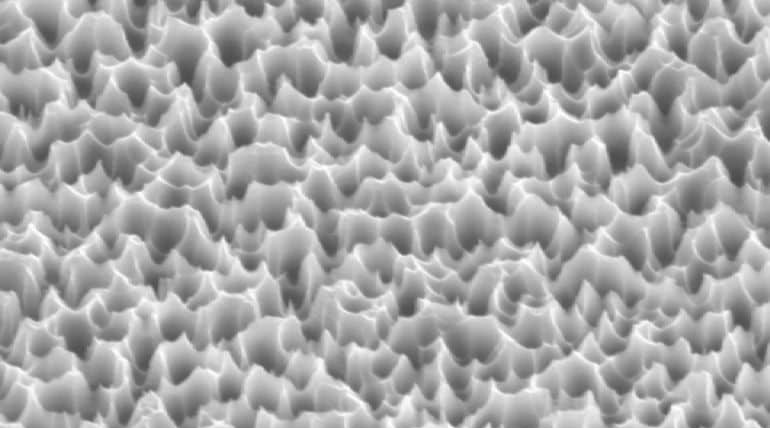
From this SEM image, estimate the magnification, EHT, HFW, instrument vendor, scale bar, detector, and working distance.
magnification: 40 K X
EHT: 5 kV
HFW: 7.534 µm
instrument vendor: Zeiss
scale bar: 1000 nm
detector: SE2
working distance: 22.9 mm